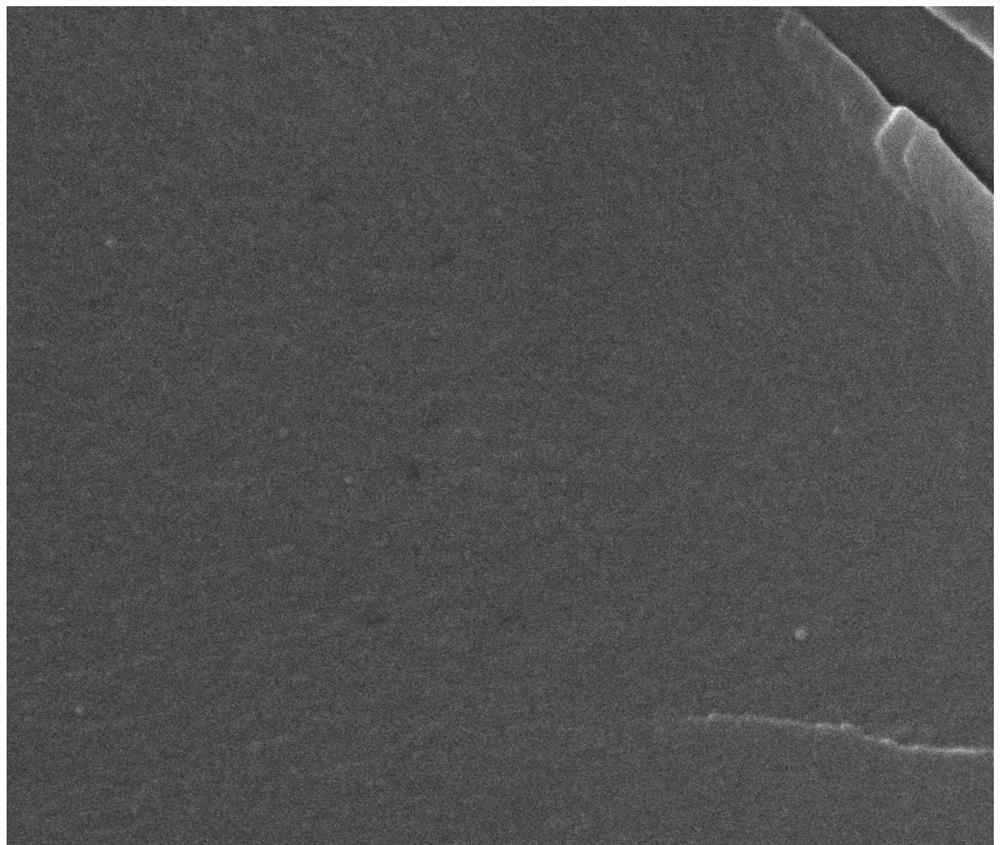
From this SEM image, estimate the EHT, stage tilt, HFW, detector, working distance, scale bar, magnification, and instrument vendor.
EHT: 20 kV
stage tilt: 0°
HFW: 5.97 µm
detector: ETD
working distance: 12.8 mm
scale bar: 2000 nm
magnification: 50 K X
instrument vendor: FEI